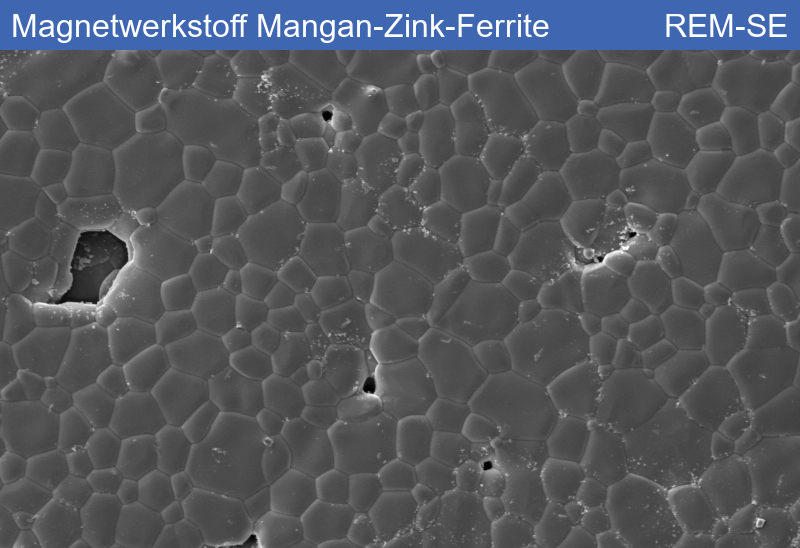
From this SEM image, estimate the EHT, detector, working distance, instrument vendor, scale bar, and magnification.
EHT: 15 kV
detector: SE1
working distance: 12.82 mm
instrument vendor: Zeiss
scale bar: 20000 nm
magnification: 0.5 K X